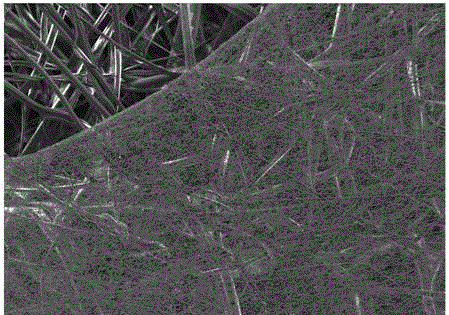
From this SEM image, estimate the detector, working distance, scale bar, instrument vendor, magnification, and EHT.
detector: SE2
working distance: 9.1 mm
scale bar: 20000 nm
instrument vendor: Zeiss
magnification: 0.5 K X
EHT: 2 kV